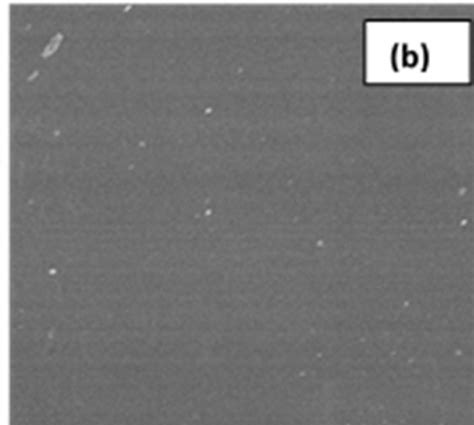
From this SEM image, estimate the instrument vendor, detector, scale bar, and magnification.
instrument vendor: Hitachi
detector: SE(UL)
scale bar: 10000 nm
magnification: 5 K X